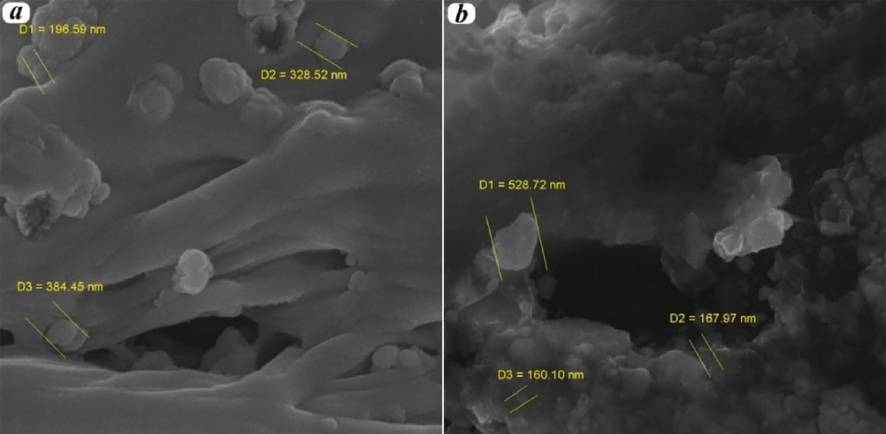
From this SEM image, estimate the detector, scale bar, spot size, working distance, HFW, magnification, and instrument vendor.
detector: InBeam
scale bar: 1000 nm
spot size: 7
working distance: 7.05 mm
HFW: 5.19 µm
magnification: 40 K X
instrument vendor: Tescan